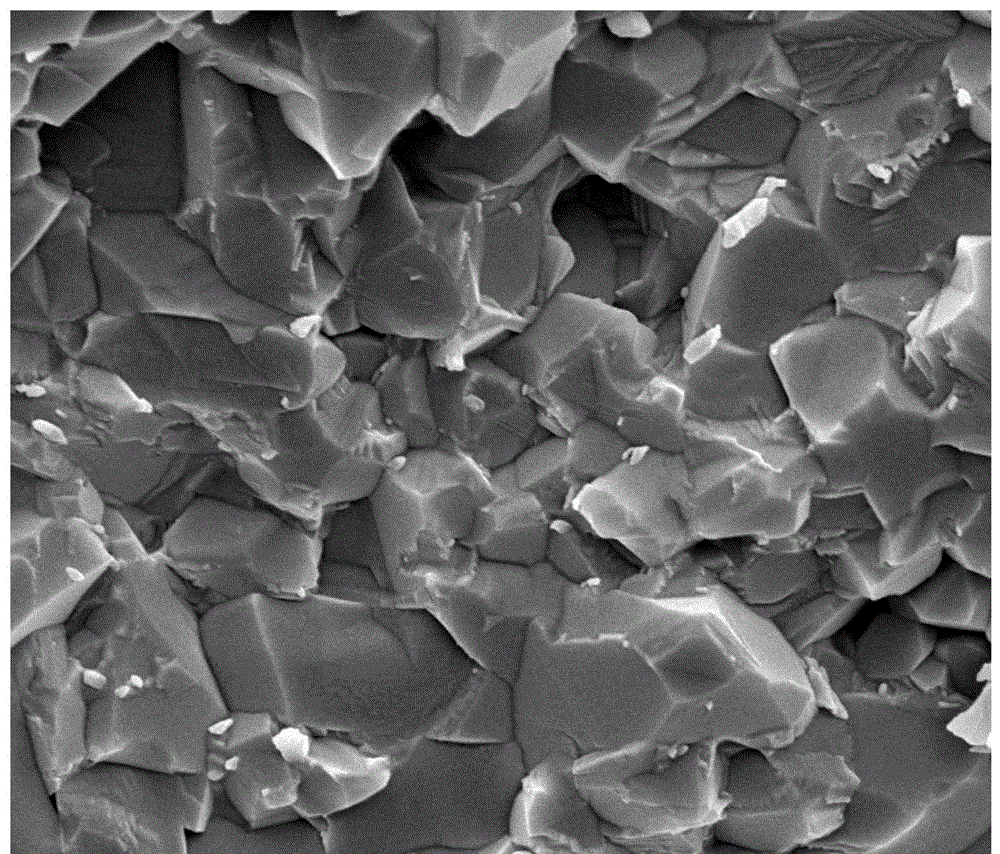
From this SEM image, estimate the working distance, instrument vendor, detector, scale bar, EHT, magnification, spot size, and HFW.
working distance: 6.9 mm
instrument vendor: FEI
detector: ETD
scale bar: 2000 nm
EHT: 8 kV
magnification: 20 K X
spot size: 3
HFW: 7.46 µm